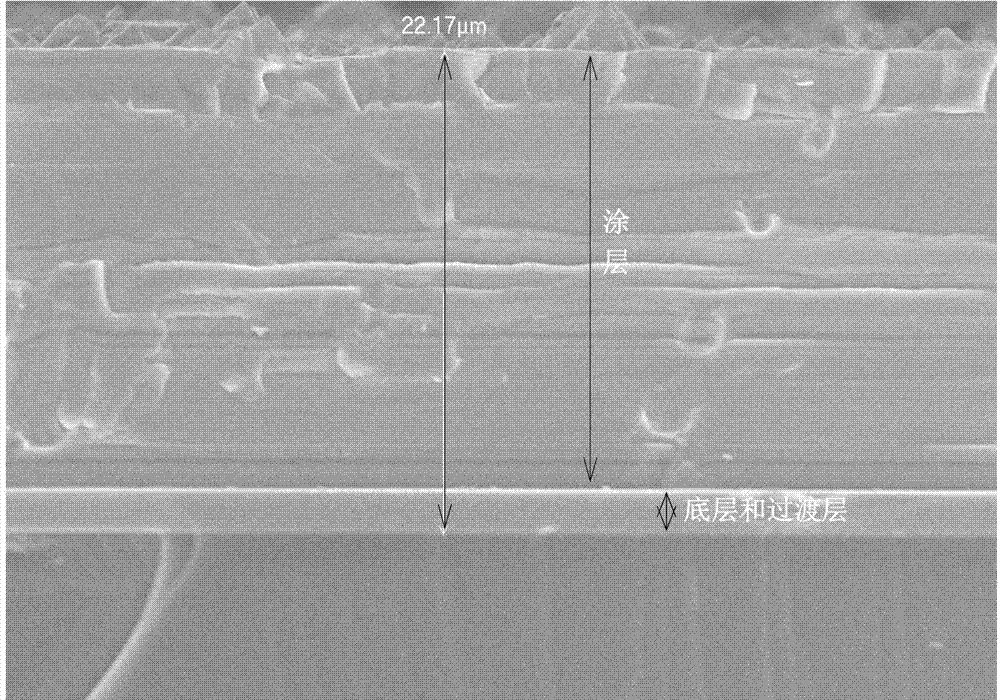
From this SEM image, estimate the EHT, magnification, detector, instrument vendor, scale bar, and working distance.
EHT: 10 kV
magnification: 2 K X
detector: SEI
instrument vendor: JEOL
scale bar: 10000 nm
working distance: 7.9 mm